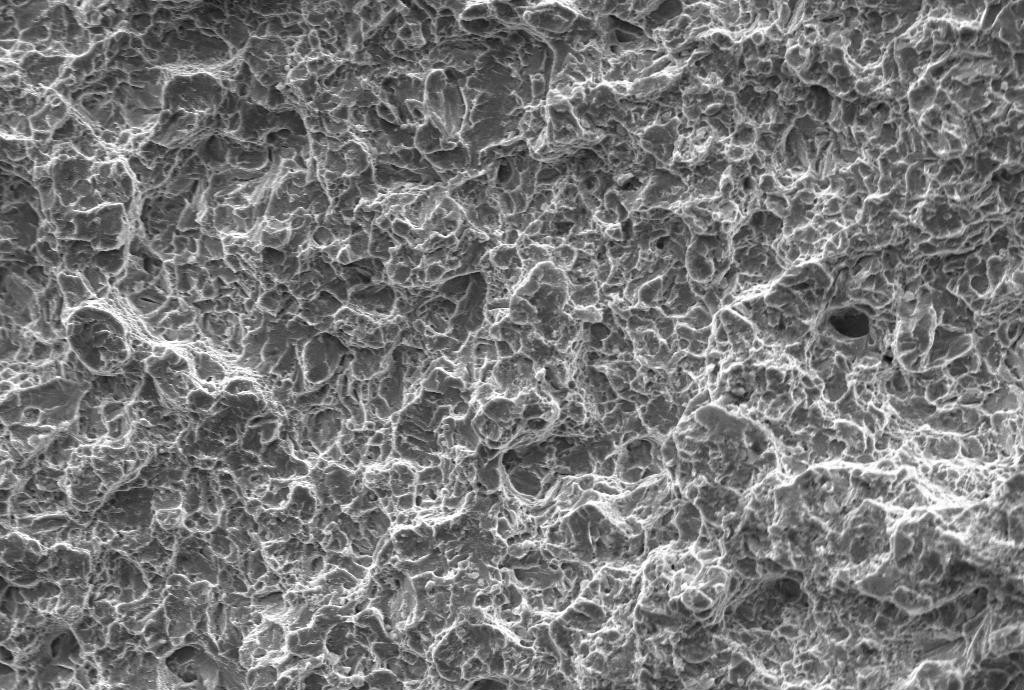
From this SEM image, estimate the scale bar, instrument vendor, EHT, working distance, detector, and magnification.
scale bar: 20000 nm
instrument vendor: Zeiss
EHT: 20 kV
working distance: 15 mm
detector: SE1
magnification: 0.5 K X